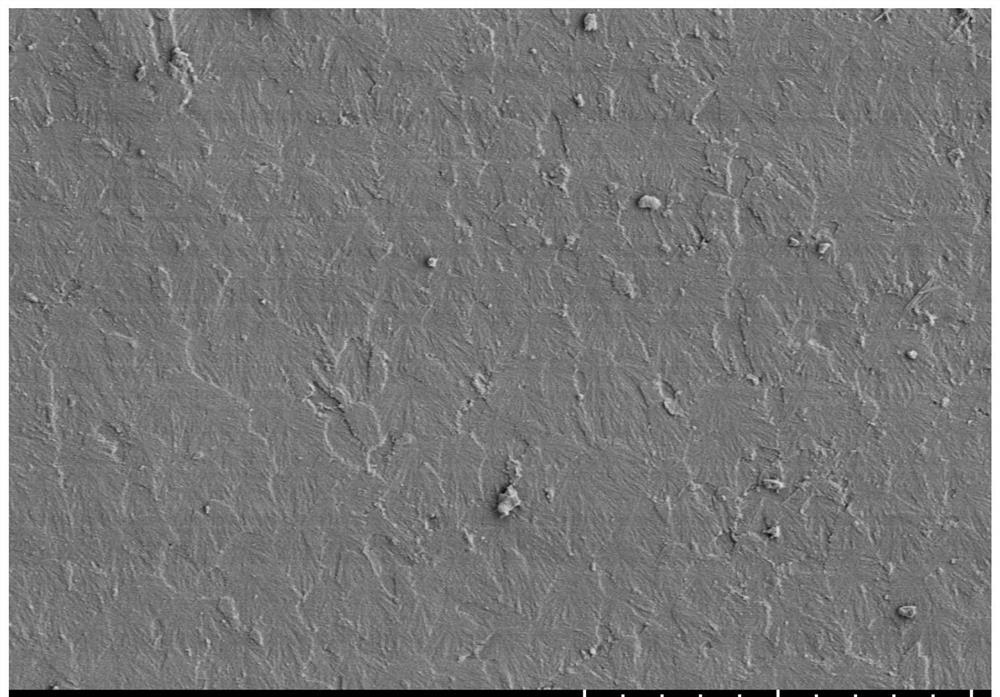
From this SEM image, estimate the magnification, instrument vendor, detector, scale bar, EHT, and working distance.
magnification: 1 K X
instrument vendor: Hitachi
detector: SE(L)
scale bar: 50000 nm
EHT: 3 kV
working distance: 12.9 mm